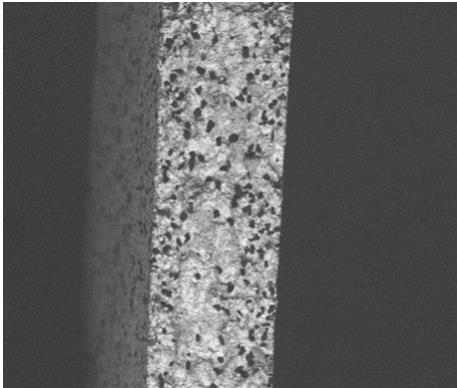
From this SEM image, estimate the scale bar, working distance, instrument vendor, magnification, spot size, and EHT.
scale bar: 500000 nm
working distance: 13.1 mm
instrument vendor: FEI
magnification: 0.2 K X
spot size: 4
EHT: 20 kV